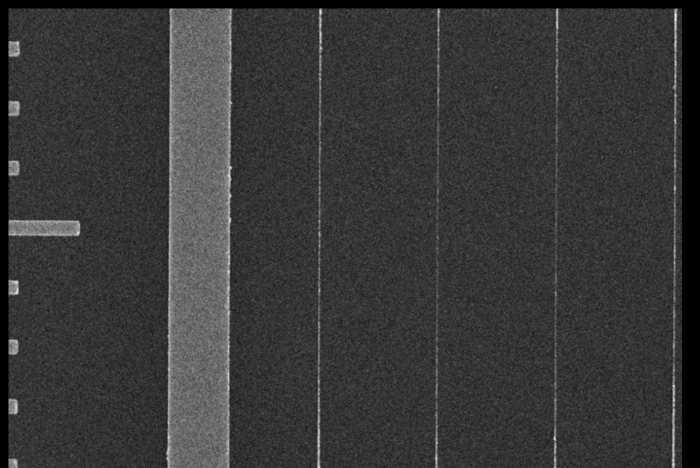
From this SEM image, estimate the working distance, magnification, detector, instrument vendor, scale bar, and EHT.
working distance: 10.9 mm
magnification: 10 K X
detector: InLens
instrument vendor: Zeiss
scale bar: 1000 nm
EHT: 20 kV